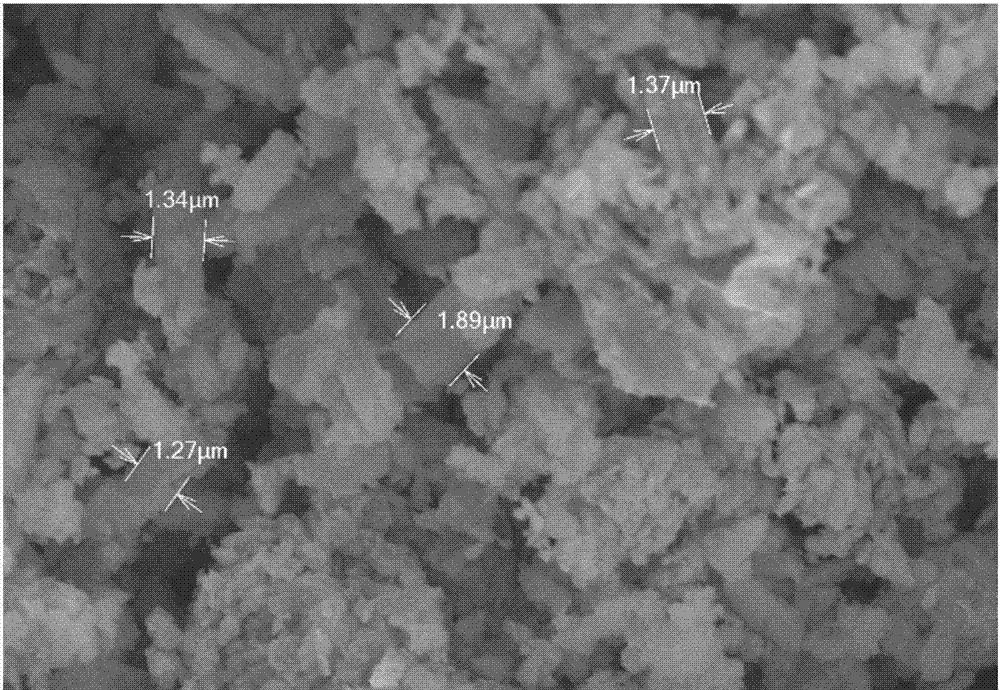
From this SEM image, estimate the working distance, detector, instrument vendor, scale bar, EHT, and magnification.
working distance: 7 mm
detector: SE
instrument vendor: Hitachi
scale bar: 10000 nm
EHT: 20 kV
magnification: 5 K X